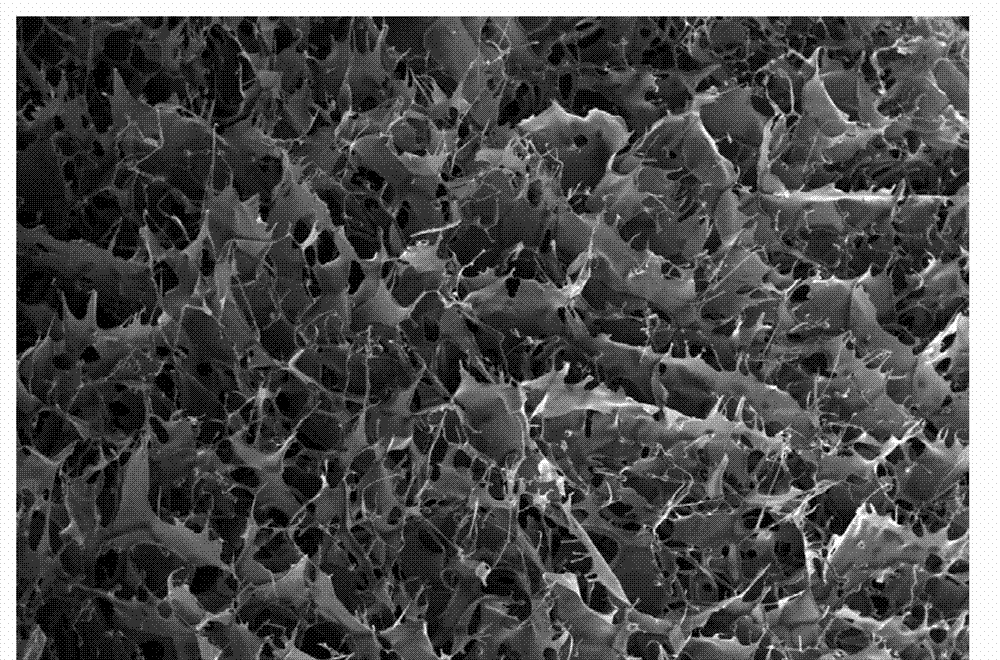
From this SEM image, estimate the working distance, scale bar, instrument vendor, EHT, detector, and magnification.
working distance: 23.1 mm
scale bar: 1000000 nm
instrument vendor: Hitachi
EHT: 20 kV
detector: SE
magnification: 0.03 K X